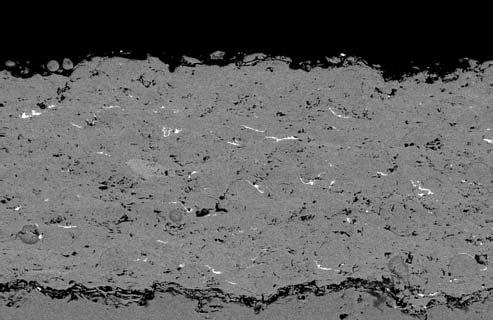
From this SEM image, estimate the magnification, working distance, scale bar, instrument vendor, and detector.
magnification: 0.15 K X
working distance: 12.5 mm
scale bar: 100000 nm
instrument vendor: Zeiss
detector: CZ BSD